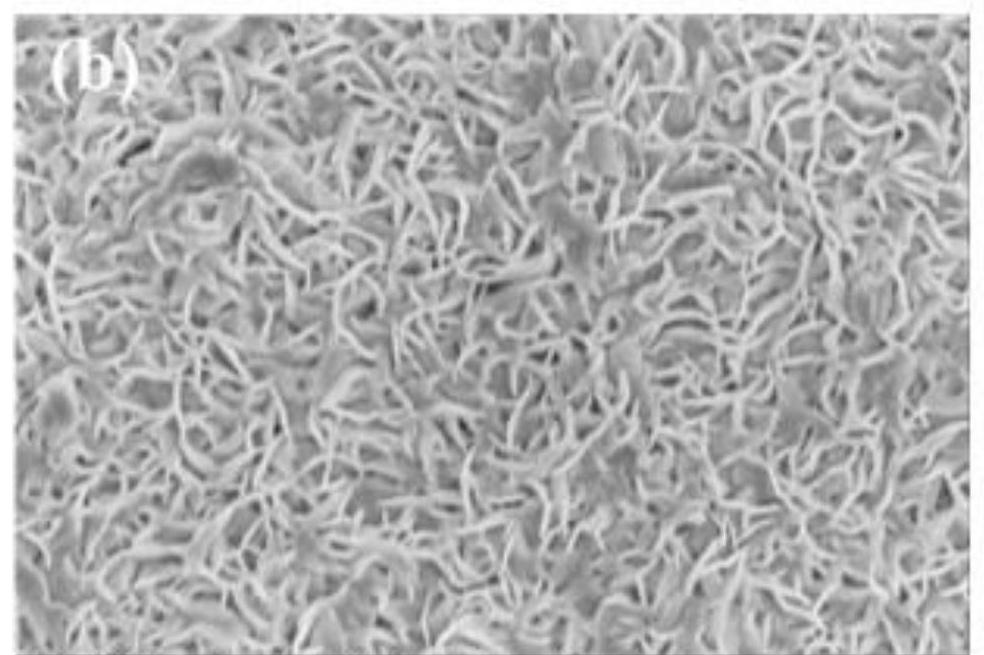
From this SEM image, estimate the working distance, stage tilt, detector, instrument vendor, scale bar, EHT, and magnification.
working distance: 5 mm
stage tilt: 0°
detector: TLD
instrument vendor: FEI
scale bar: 1000 nm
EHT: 2 kV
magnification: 100 K X